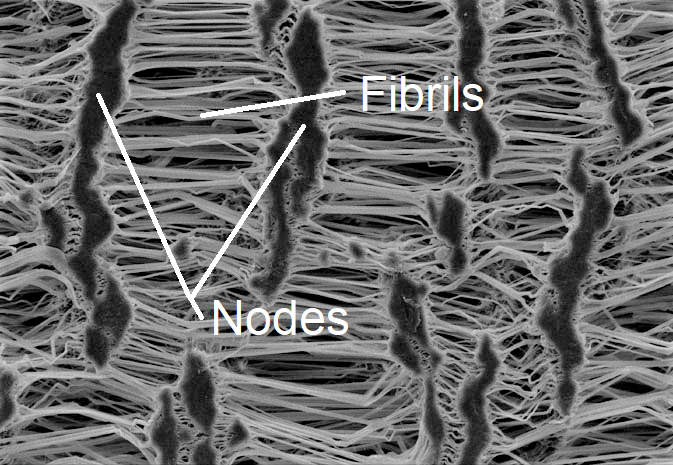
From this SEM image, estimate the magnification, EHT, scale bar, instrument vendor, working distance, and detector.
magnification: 1 K X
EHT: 10 kV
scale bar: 20000 nm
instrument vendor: Tescan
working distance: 25 mm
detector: SE